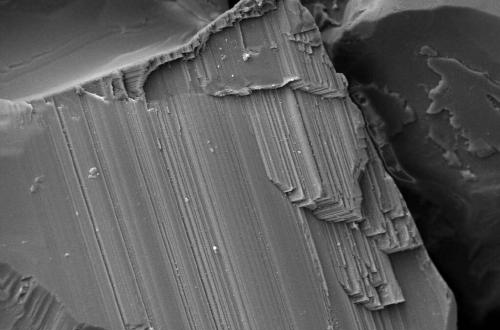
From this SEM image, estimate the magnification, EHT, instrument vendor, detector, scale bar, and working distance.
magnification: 0.5 K X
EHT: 20 kV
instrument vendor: Zeiss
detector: SE1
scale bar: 10000 nm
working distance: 8.5 mm